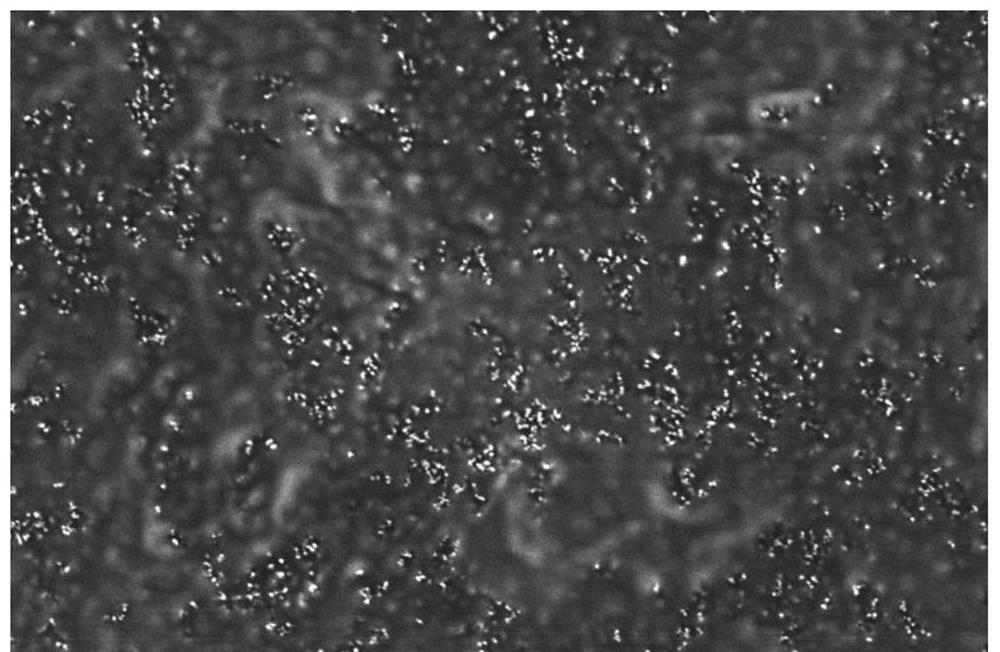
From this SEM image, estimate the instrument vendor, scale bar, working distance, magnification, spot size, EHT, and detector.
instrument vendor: FEI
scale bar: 5000 nm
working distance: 5.4 mm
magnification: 24 K X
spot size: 3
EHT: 2 kV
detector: CBS